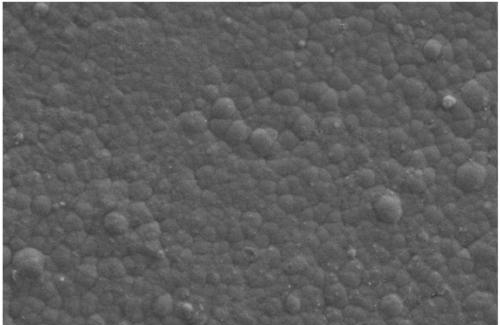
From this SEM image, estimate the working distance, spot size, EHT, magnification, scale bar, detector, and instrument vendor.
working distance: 4.8 mm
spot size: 4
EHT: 5 kV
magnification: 20 K X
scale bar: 2000 nm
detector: SE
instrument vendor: FEI